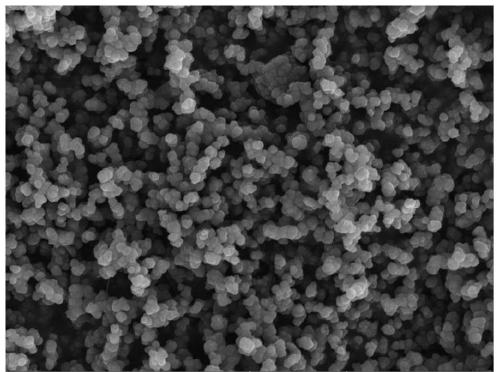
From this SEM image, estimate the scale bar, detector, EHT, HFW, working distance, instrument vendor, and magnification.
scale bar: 5000 nm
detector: SE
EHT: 8 kV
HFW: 25.3 µm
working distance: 11.42 mm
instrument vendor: Tescan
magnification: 10 K X